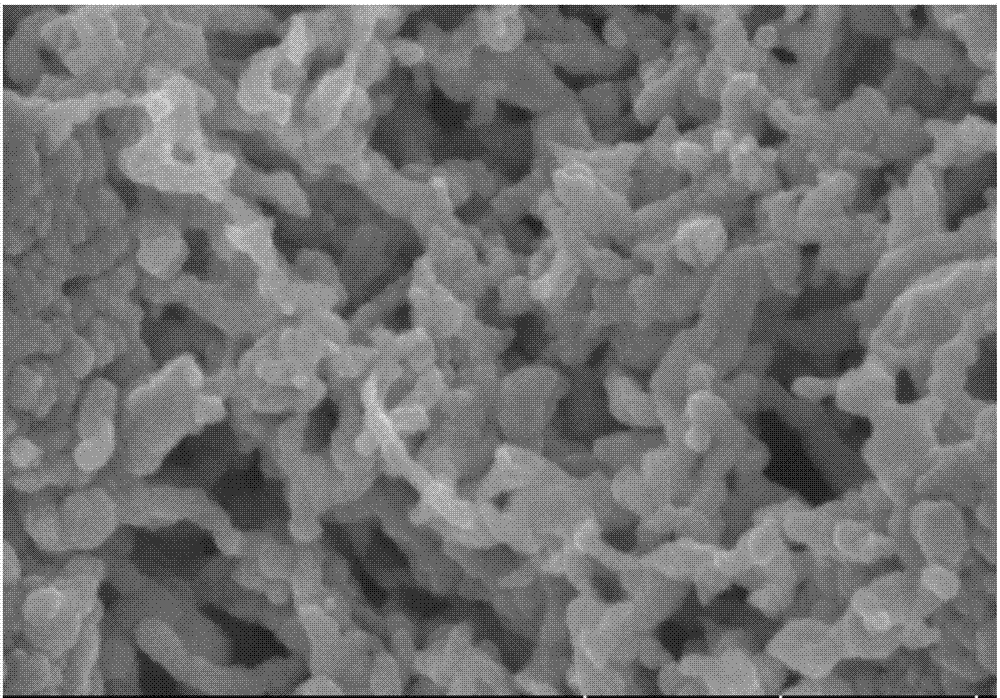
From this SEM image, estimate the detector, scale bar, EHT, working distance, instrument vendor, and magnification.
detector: SE(U)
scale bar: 500 nm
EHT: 5 kV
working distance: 9.5 mm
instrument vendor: Hitachi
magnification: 100 K X